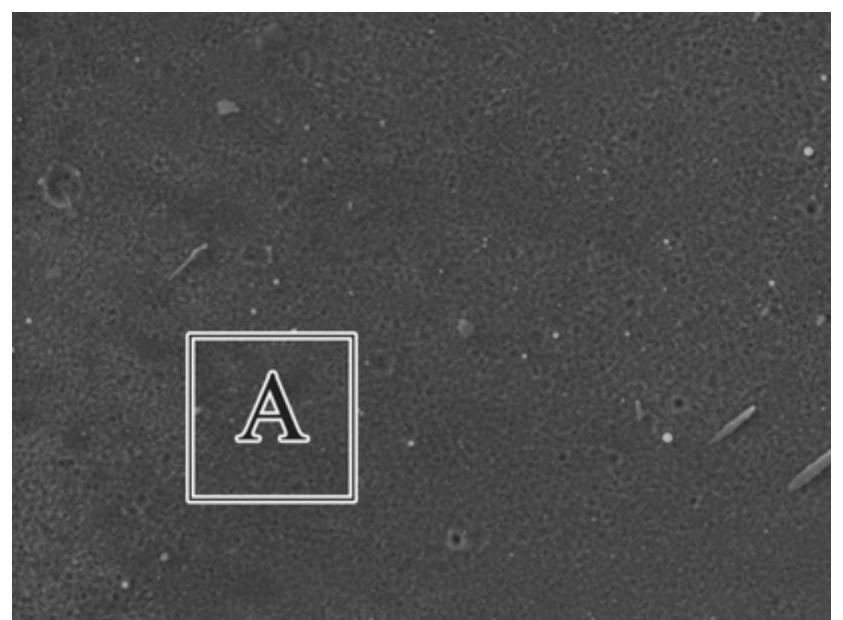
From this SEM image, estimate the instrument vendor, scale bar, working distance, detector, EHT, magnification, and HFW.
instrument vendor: Tescan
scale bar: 10000 nm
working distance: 9.04 mm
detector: SE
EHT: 20 kV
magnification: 5 K X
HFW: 55.4 µm